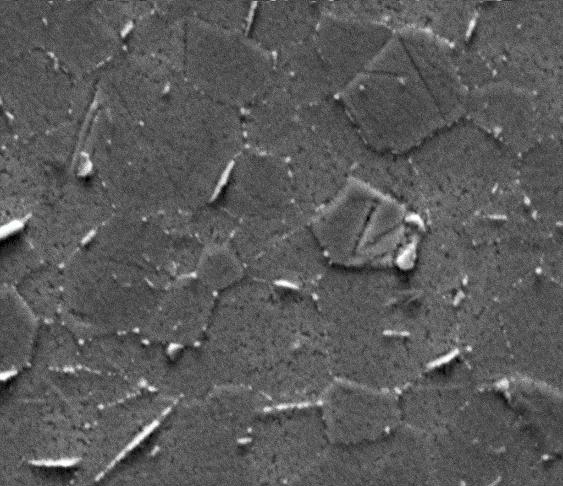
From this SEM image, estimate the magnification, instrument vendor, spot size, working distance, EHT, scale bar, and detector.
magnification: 1 K X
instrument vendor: FEI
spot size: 5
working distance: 10.1 mm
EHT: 30 kV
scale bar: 50000 nm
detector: Mix